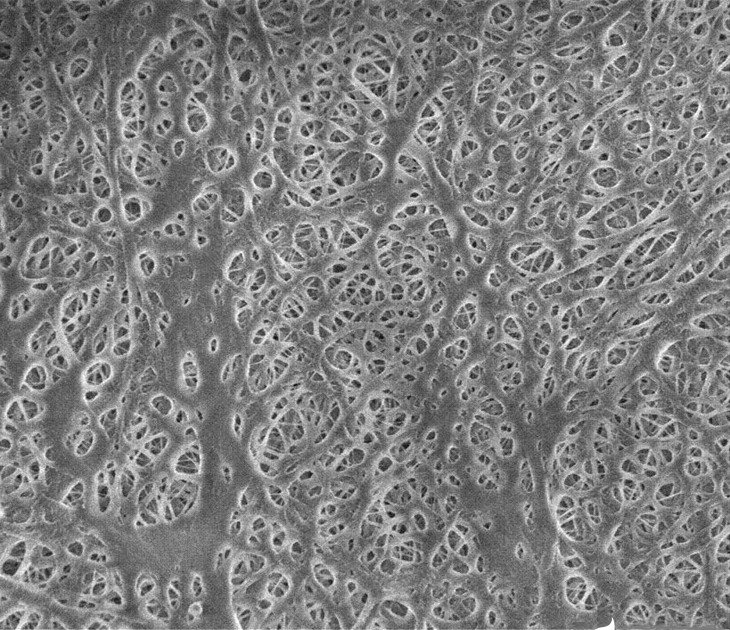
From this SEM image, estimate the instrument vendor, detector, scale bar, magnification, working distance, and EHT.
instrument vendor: FEI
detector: TLD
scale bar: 3000 nm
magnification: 20 K X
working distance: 1.5 mm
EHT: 1.5 kV